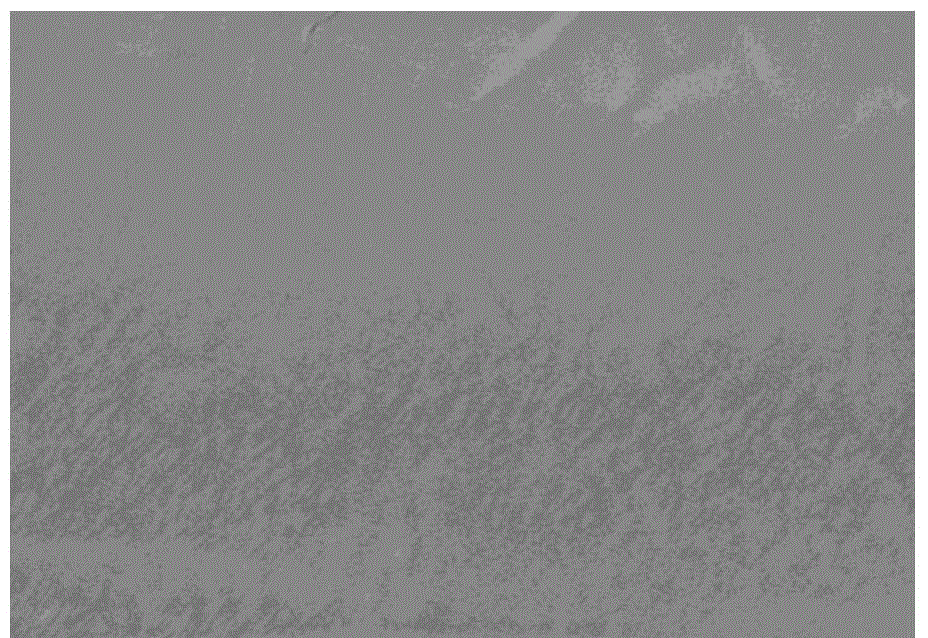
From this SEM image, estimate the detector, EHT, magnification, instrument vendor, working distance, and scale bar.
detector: SE(M)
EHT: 3 kV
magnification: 10 K X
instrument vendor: Hitachi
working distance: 8.8 mm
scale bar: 5000 nm